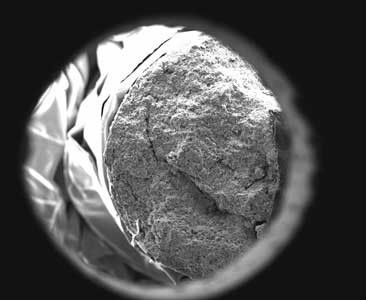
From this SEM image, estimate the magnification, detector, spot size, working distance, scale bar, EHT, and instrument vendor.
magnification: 0.03 K X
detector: SE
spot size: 4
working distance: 11.3 mm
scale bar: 2000000 nm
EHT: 25 kV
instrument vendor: FEI